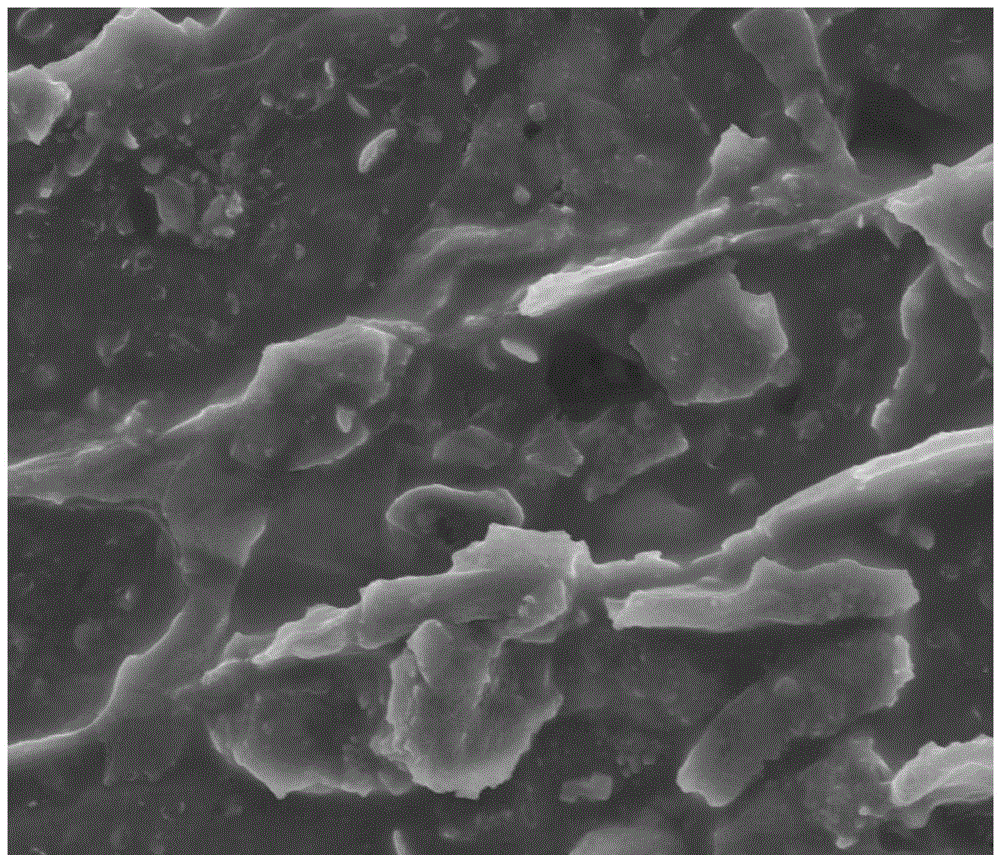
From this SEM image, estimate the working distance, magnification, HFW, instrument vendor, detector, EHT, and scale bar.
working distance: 9.8 mm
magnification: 10 K X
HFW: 29.8 µm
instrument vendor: FEI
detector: ETD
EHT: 10 kV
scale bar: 10000 nm